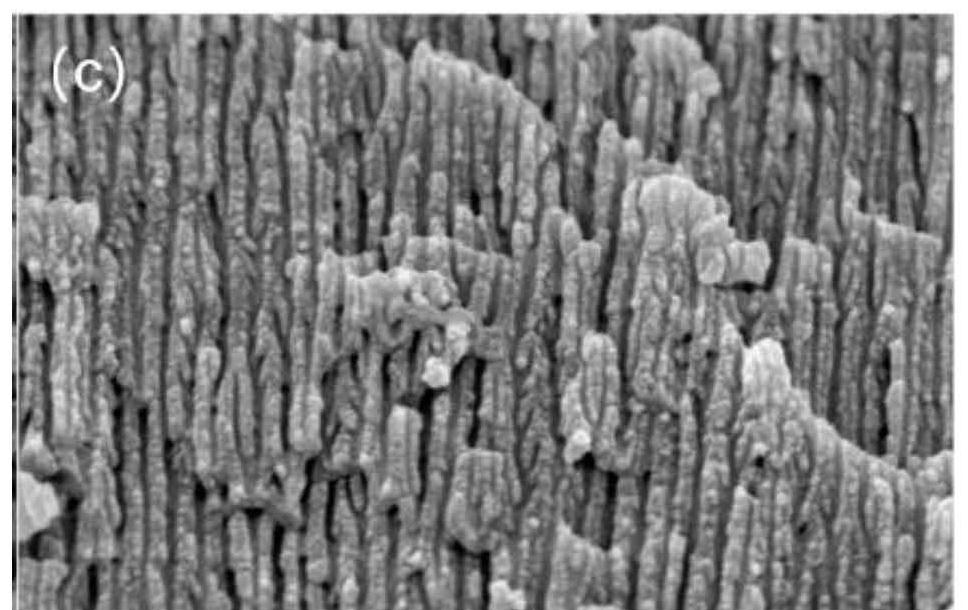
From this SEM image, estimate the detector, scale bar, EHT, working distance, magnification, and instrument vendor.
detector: SE(M)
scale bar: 500 nm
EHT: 3 kV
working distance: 3.9 mm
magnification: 100 K X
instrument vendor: Hitachi